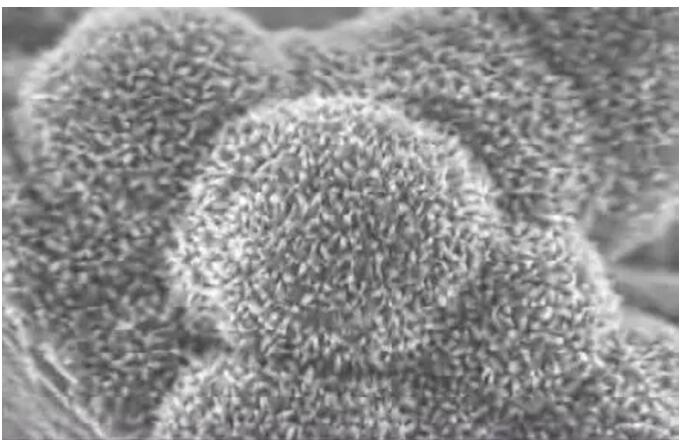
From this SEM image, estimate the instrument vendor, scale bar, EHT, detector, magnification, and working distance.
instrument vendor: JEOL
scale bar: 100 nm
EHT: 5 kV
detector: SEI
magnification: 50 K X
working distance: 3 mm